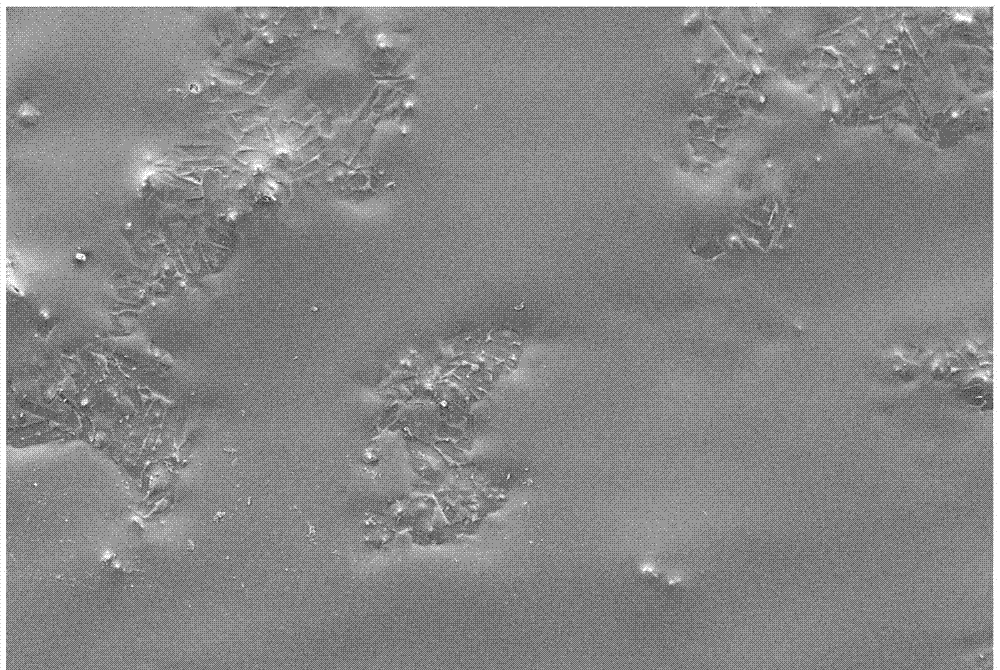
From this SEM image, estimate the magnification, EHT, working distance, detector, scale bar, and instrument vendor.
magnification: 0.5 K X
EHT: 5 kV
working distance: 5.4 mm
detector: SE2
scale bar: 10000 nm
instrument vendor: Zeiss